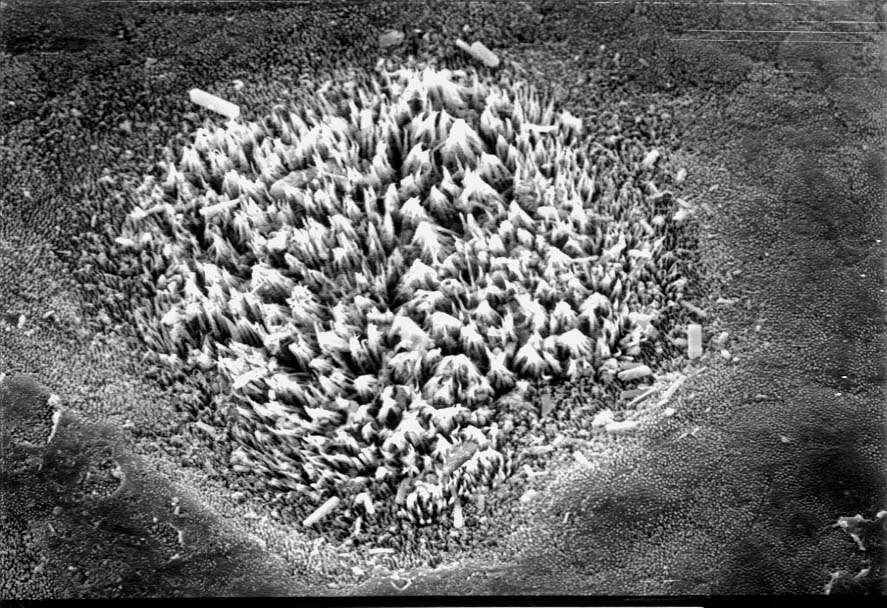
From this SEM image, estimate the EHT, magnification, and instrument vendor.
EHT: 20 kV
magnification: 0.68 K X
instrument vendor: JEOL